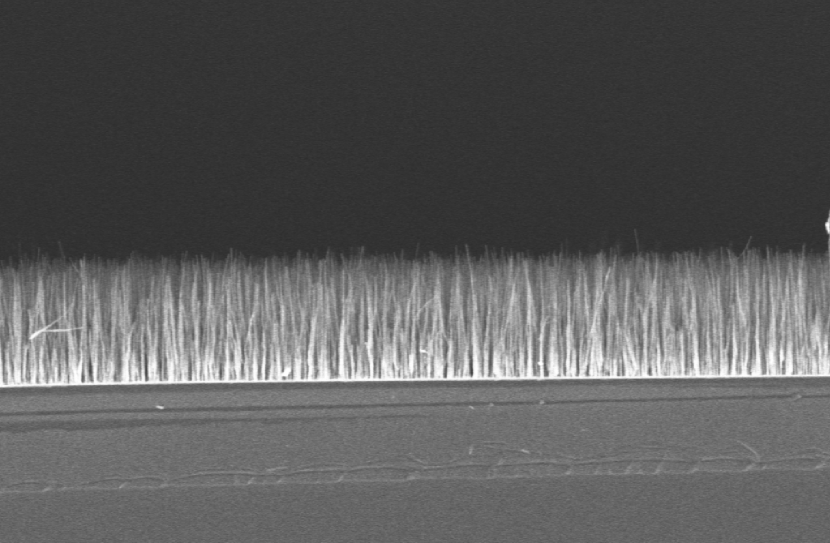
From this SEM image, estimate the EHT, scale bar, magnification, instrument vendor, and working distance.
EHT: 5 kV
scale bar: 10000 nm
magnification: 4.53 K X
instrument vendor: Zeiss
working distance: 3 mm